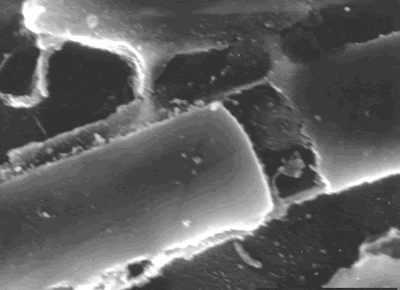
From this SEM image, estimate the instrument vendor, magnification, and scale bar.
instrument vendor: JEOL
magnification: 6 K X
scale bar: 5000 nm